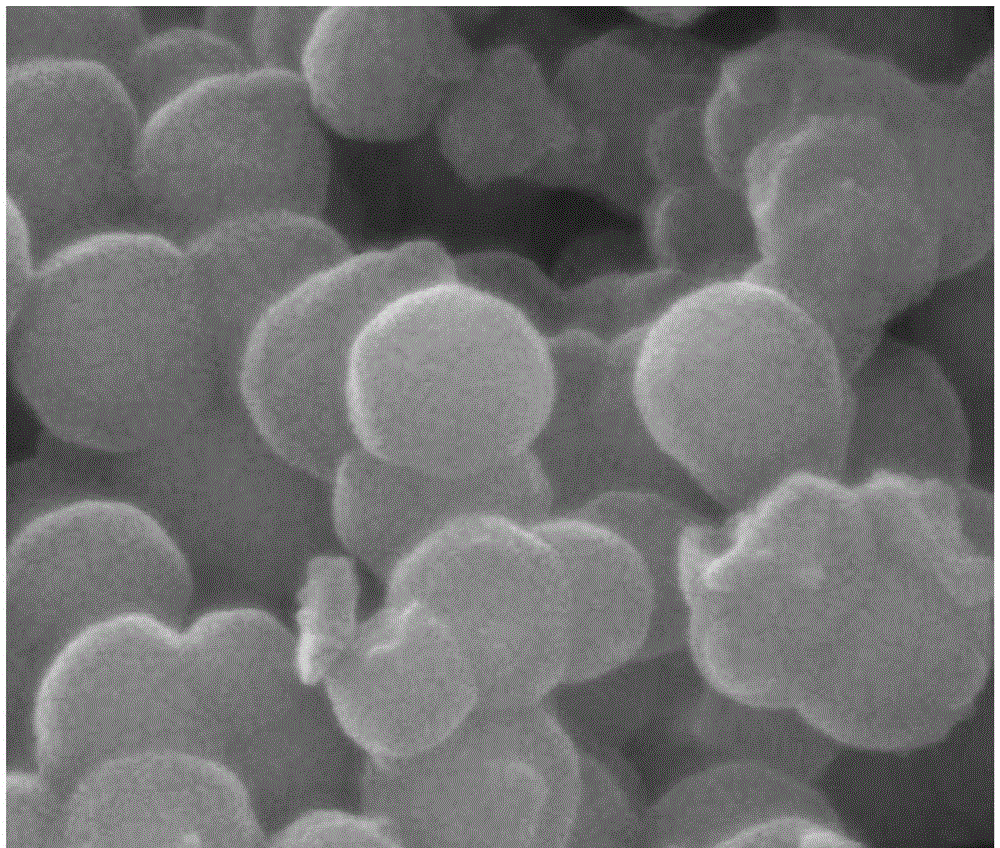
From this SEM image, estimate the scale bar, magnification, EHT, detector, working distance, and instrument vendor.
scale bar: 500 nm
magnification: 200 K X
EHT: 30 kV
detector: SE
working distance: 10.3 mm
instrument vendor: FEI